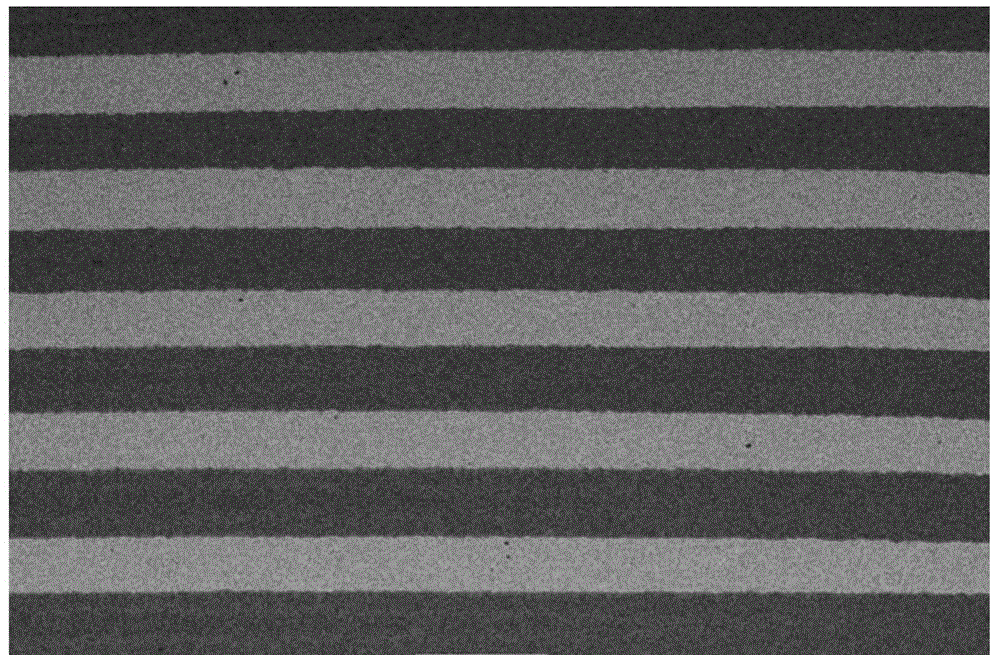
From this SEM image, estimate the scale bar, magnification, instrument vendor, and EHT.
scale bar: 1000000 nm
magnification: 0.017 K X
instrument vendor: JEOL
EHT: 20 kV